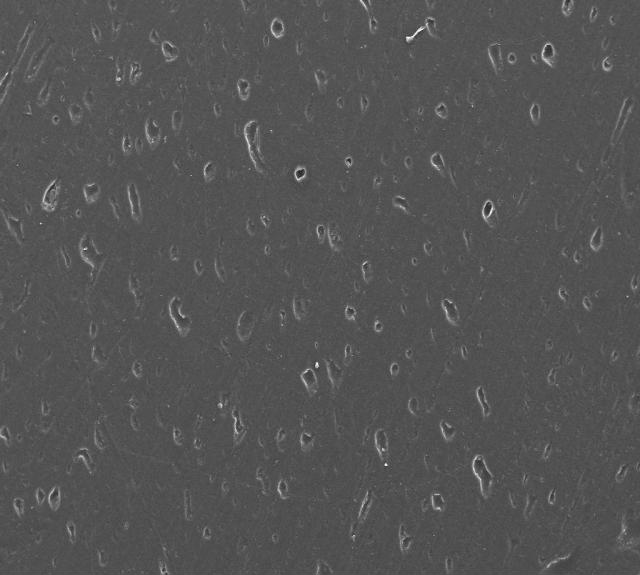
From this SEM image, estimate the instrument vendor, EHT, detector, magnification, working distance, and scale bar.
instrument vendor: Zeiss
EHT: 20 kV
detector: SE1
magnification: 0.2 K X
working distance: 15.5 mm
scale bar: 200000 nm